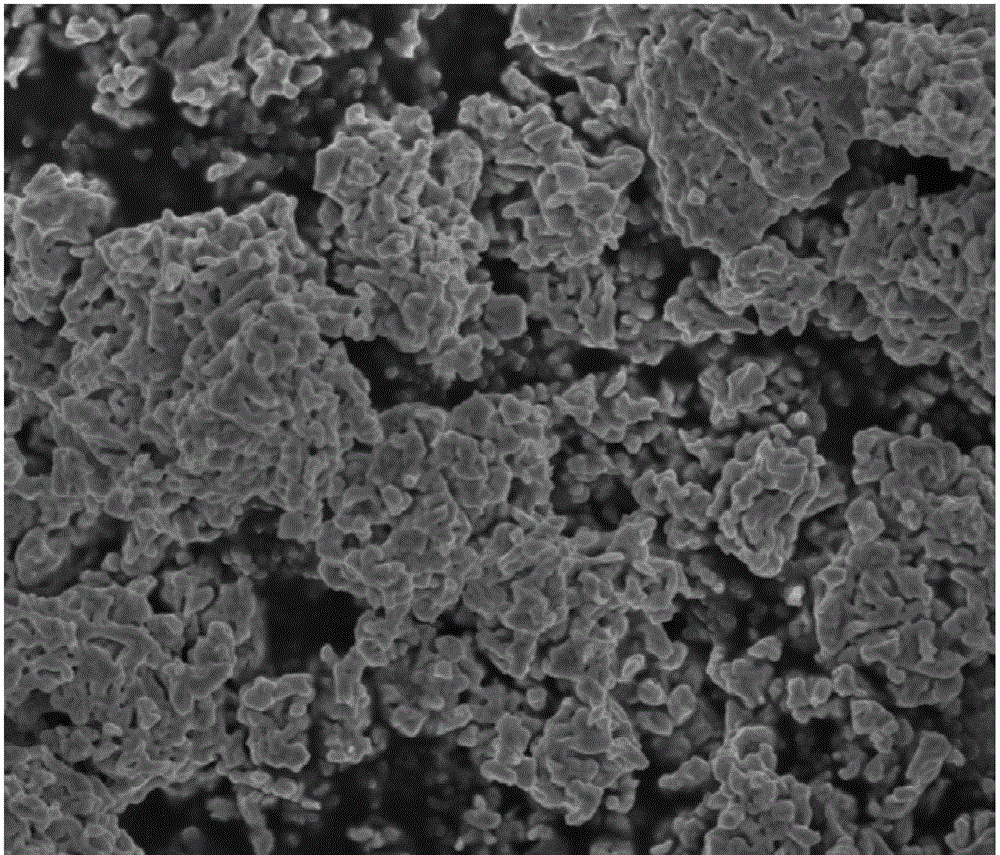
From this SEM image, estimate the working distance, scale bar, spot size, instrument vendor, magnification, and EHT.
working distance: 6.6 mm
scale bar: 1000 nm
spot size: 3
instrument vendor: FEI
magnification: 60 K X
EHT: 3 kV